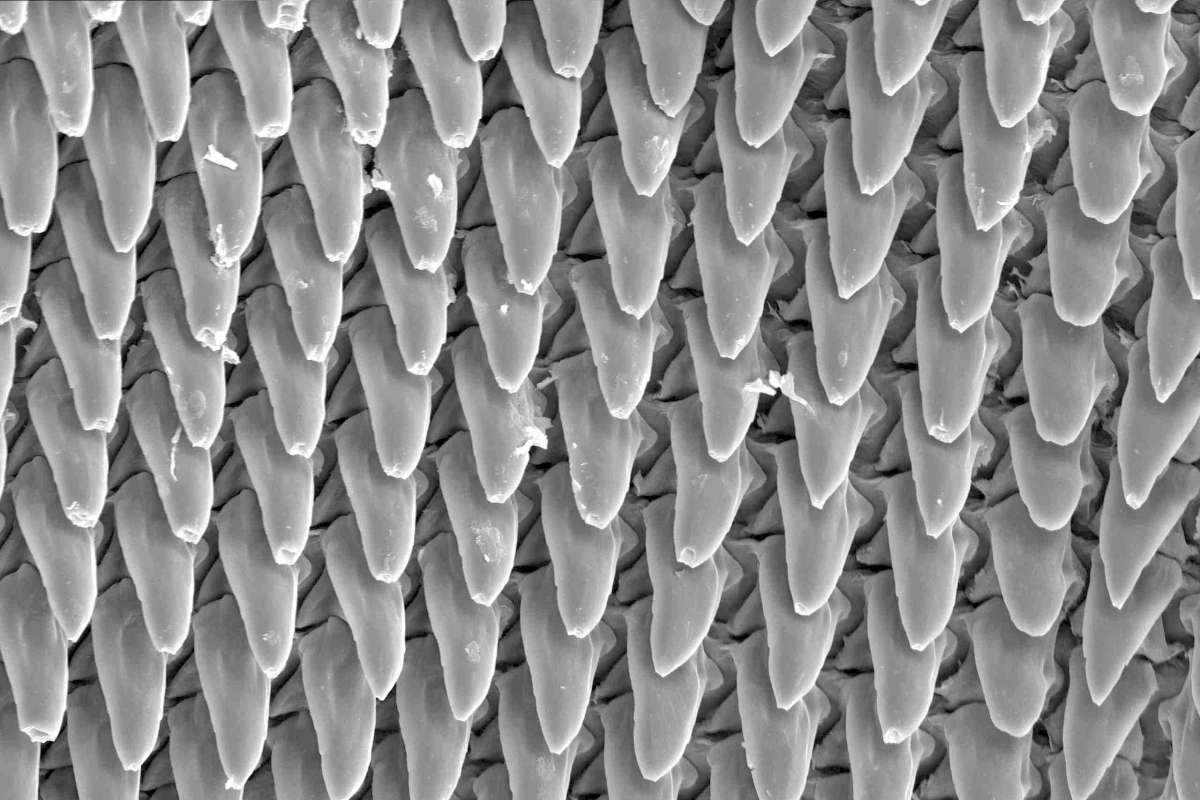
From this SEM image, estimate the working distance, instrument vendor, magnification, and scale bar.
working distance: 16.4 mm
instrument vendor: Hitachi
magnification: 0.5 K X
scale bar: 100000 nm